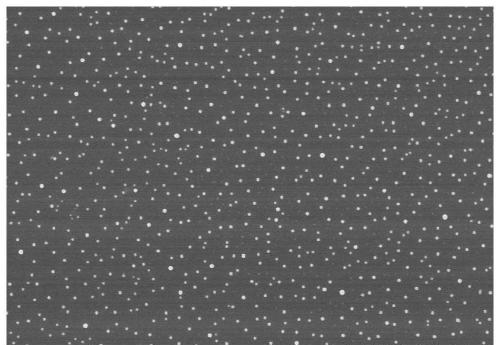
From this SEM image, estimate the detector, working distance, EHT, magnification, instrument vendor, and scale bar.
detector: SE(U,LA0)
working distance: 8.3 mm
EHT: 3 kV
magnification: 20 K X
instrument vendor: Hitachi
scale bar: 2000 nm